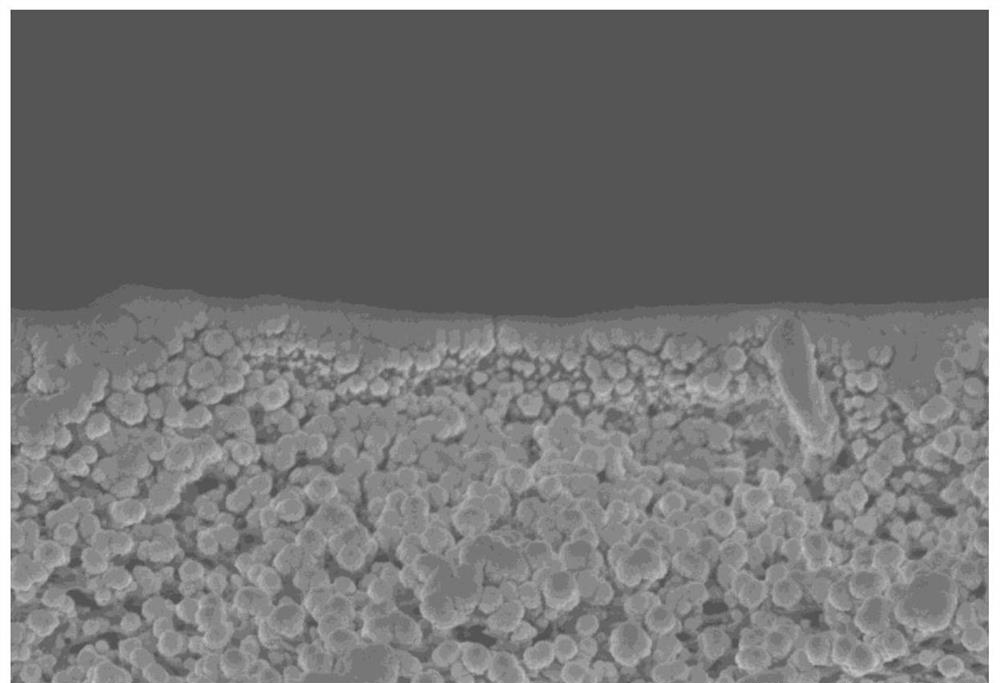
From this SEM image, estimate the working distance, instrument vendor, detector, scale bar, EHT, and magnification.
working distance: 8.7 mm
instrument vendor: Hitachi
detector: SE(M)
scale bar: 2000 nm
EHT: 3 kV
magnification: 20 K X